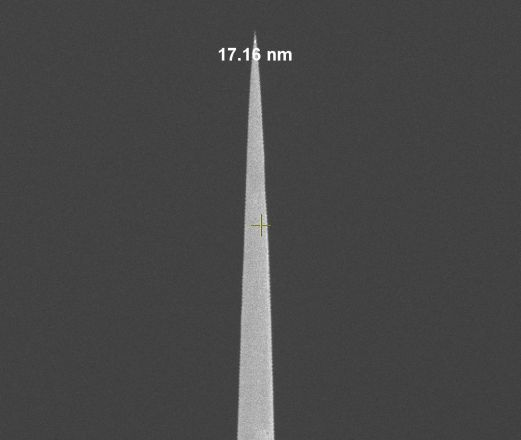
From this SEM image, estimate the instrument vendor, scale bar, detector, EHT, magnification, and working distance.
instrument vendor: FEI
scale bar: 2000 nm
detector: TLD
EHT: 3 kV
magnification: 60 K X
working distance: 5.1 mm